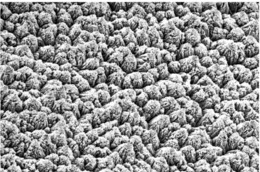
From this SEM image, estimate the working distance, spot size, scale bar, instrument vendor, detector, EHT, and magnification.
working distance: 40 mm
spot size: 50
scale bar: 10000 nm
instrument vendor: JEOL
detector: SEI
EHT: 20 kV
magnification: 1 K X